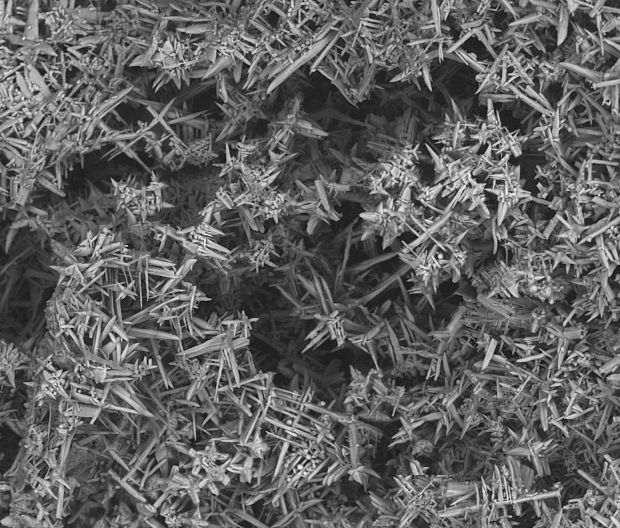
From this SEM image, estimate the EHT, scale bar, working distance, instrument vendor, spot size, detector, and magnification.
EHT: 10 kV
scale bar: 40000 nm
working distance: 9.8 mm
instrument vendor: FEI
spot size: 6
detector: BSED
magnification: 2.4 K X